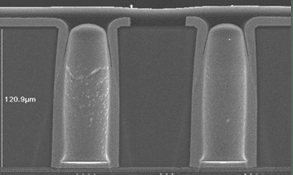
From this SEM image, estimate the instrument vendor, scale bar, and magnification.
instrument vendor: JEOL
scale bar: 66700 nm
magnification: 0.45 K X